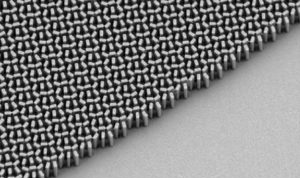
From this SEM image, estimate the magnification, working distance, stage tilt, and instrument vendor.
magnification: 22.81 K X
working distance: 6.7 mm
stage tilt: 30°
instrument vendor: Zeiss